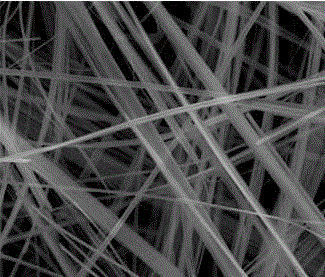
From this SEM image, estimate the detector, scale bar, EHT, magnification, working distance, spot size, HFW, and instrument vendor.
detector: ETD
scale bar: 4000 nm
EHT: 20 kV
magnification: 10 K X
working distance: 7.9 mm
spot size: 3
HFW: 14.9 µm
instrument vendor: FEI